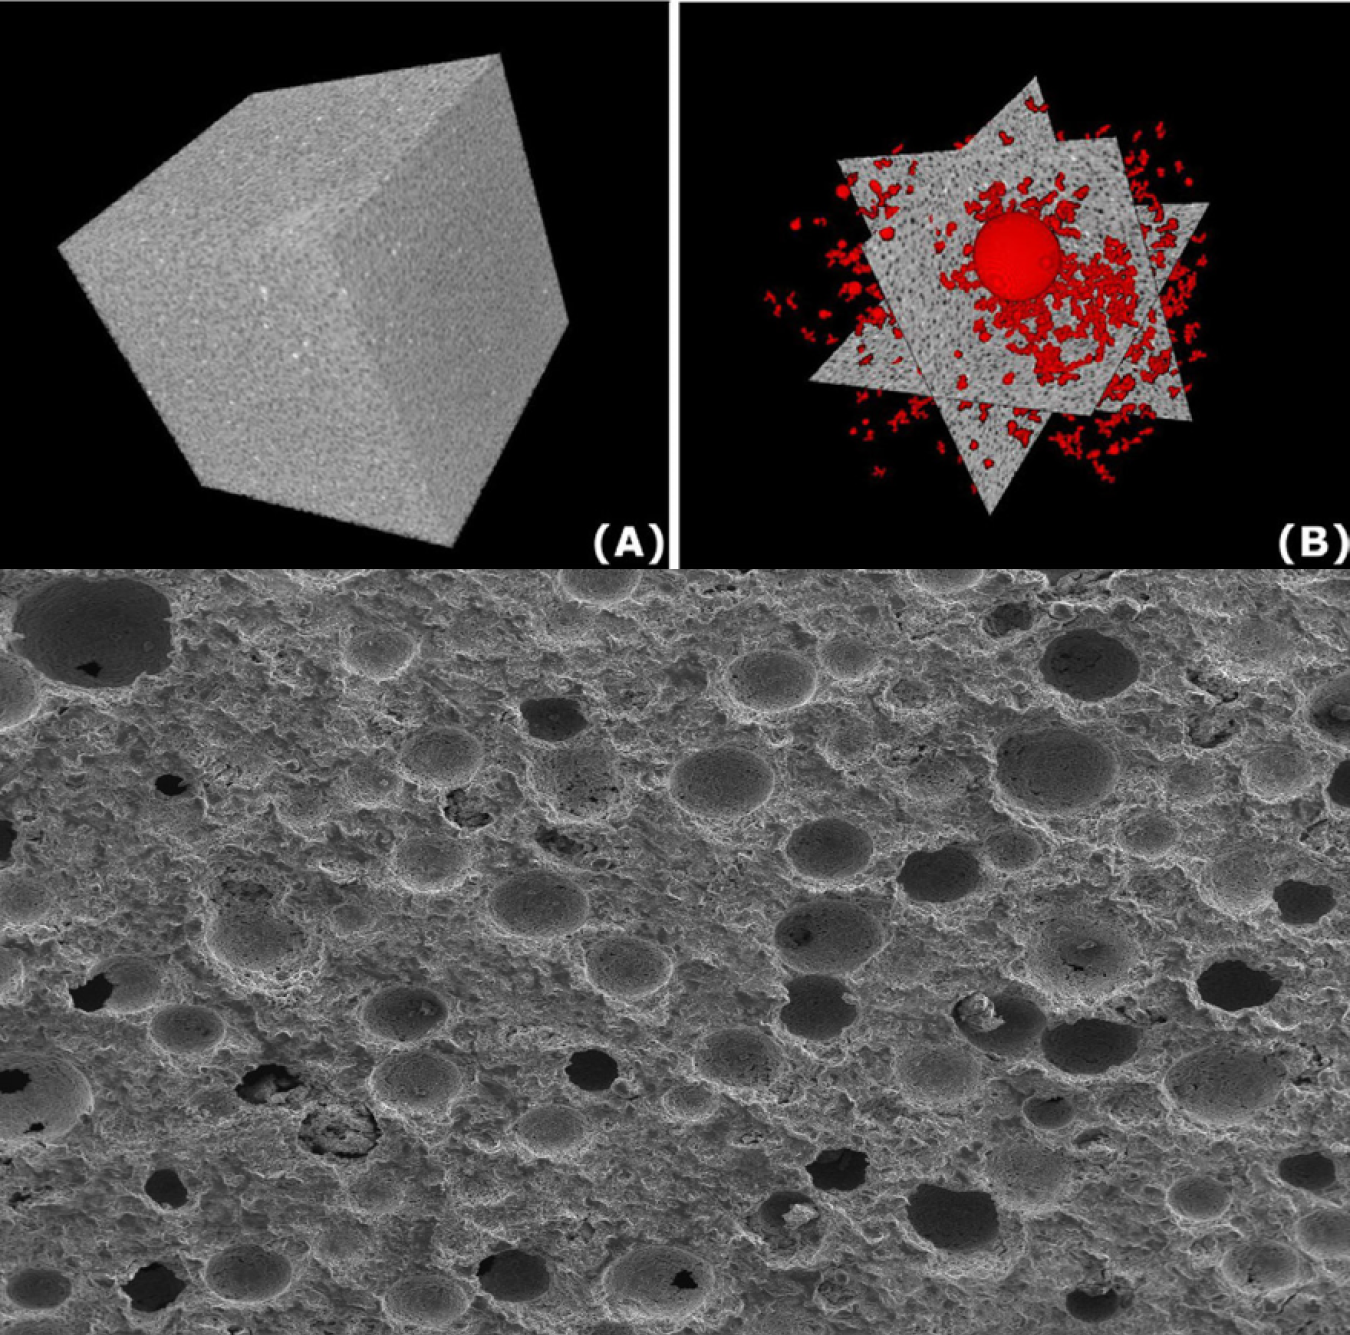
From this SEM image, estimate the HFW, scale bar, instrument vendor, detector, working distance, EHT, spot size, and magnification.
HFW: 2560 µm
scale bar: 1000000 nm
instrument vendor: FEI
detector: ETD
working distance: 10 mm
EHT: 15 kV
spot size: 3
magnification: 0.1 K X